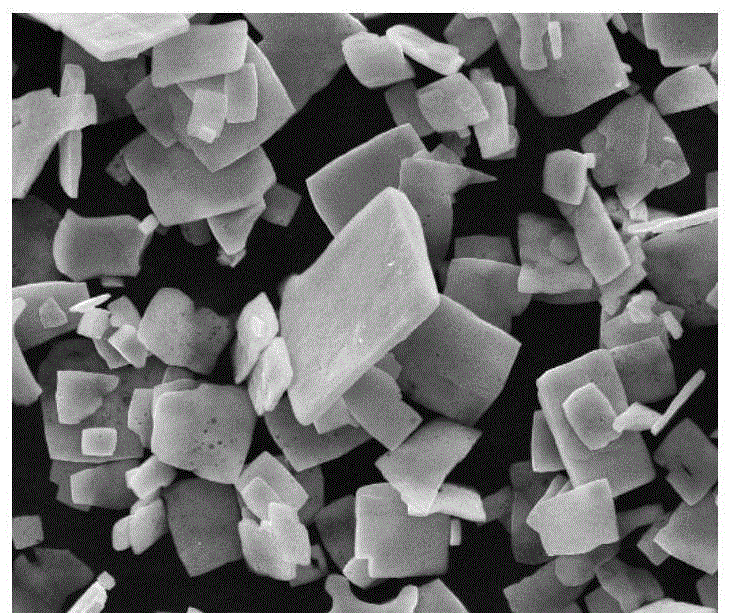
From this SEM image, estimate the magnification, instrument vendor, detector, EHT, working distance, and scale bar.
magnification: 6 K X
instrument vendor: FEI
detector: ETD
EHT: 20 kV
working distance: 11.2 mm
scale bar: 20000 nm